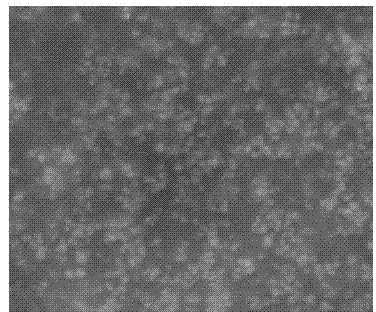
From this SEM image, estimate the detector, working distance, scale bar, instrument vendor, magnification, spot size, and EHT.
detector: SE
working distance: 9.5 mm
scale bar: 5000 nm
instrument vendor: FEI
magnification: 10 K X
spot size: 4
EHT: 20 kV